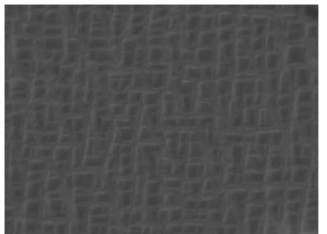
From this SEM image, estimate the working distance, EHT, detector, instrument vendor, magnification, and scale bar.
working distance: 9.96 mm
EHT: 30 kV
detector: BSE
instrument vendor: Tescan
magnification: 20 K X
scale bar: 2000 nm